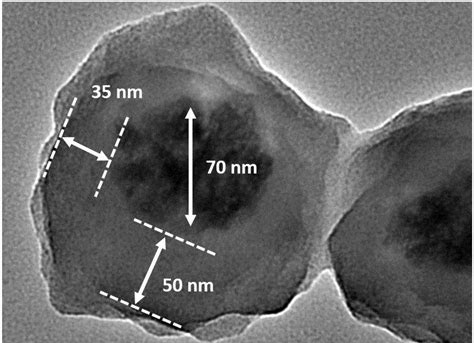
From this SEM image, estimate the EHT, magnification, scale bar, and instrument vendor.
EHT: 200 kV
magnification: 80 K X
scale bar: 50 nm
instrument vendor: JEOL